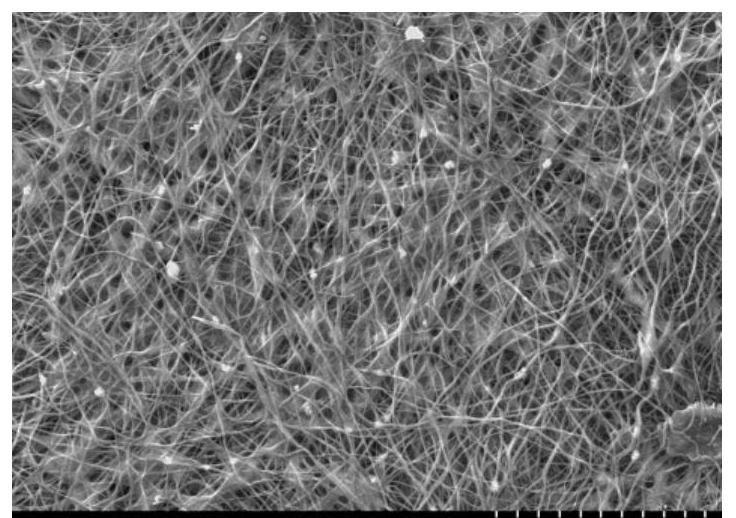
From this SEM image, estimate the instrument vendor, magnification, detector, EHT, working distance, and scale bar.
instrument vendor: Hitachi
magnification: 0.748 K X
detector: SE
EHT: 20 kV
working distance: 5.4 mm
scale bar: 50000 nm